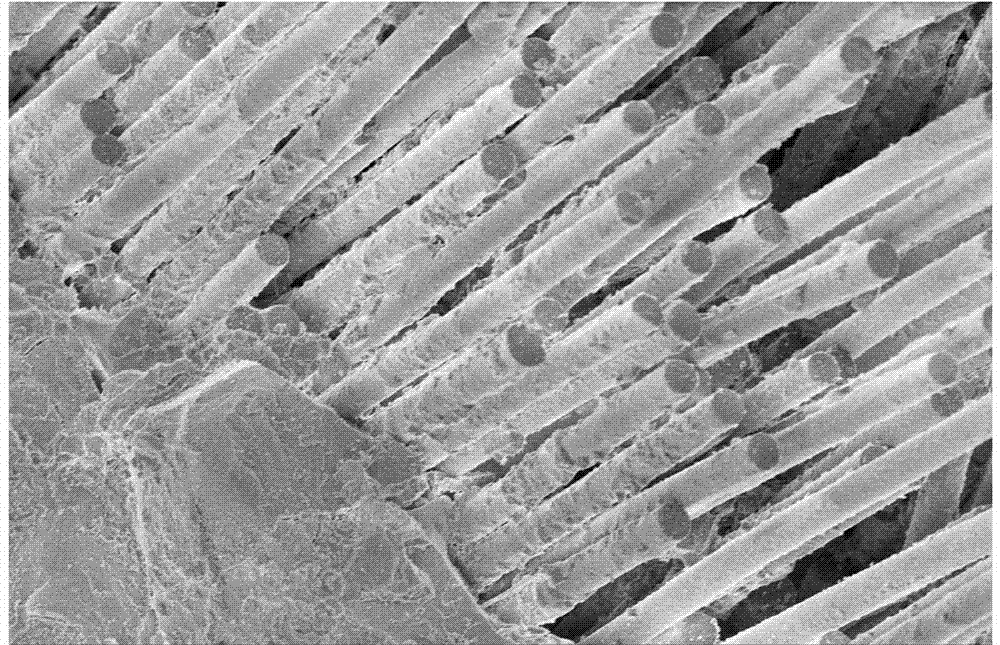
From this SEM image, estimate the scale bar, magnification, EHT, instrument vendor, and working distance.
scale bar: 100000 nm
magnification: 0.5 K X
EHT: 5 kV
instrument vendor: Hitachi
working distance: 9.6 mm